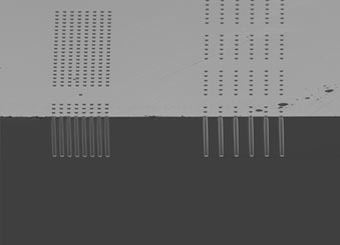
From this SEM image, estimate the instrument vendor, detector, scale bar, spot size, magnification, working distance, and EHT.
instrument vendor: FEI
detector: SE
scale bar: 100000 nm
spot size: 3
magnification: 0.274 K X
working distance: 4.9 mm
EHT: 5 kV